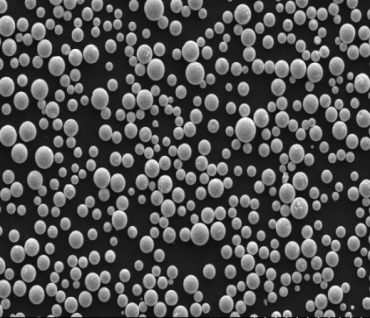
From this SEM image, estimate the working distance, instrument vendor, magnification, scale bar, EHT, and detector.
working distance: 18.6 mm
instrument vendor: Hitachi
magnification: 0.5 K X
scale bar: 200000 nm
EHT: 10 kV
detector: LM(UL)